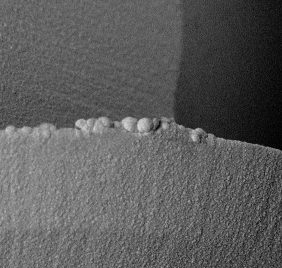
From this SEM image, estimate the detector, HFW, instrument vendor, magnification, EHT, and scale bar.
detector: BSD Full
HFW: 268 µm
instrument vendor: other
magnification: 1 K X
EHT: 15 kV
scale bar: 80000 nm